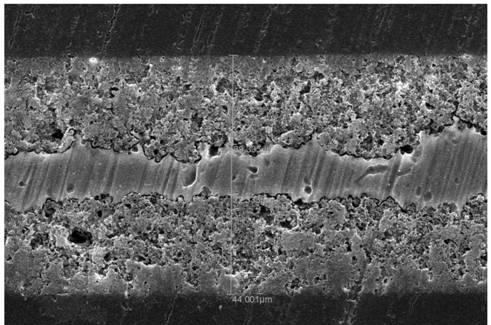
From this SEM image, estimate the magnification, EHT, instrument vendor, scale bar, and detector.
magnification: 0.6 K X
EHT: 30 kV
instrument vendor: JEOL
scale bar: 20000 nm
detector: SEI